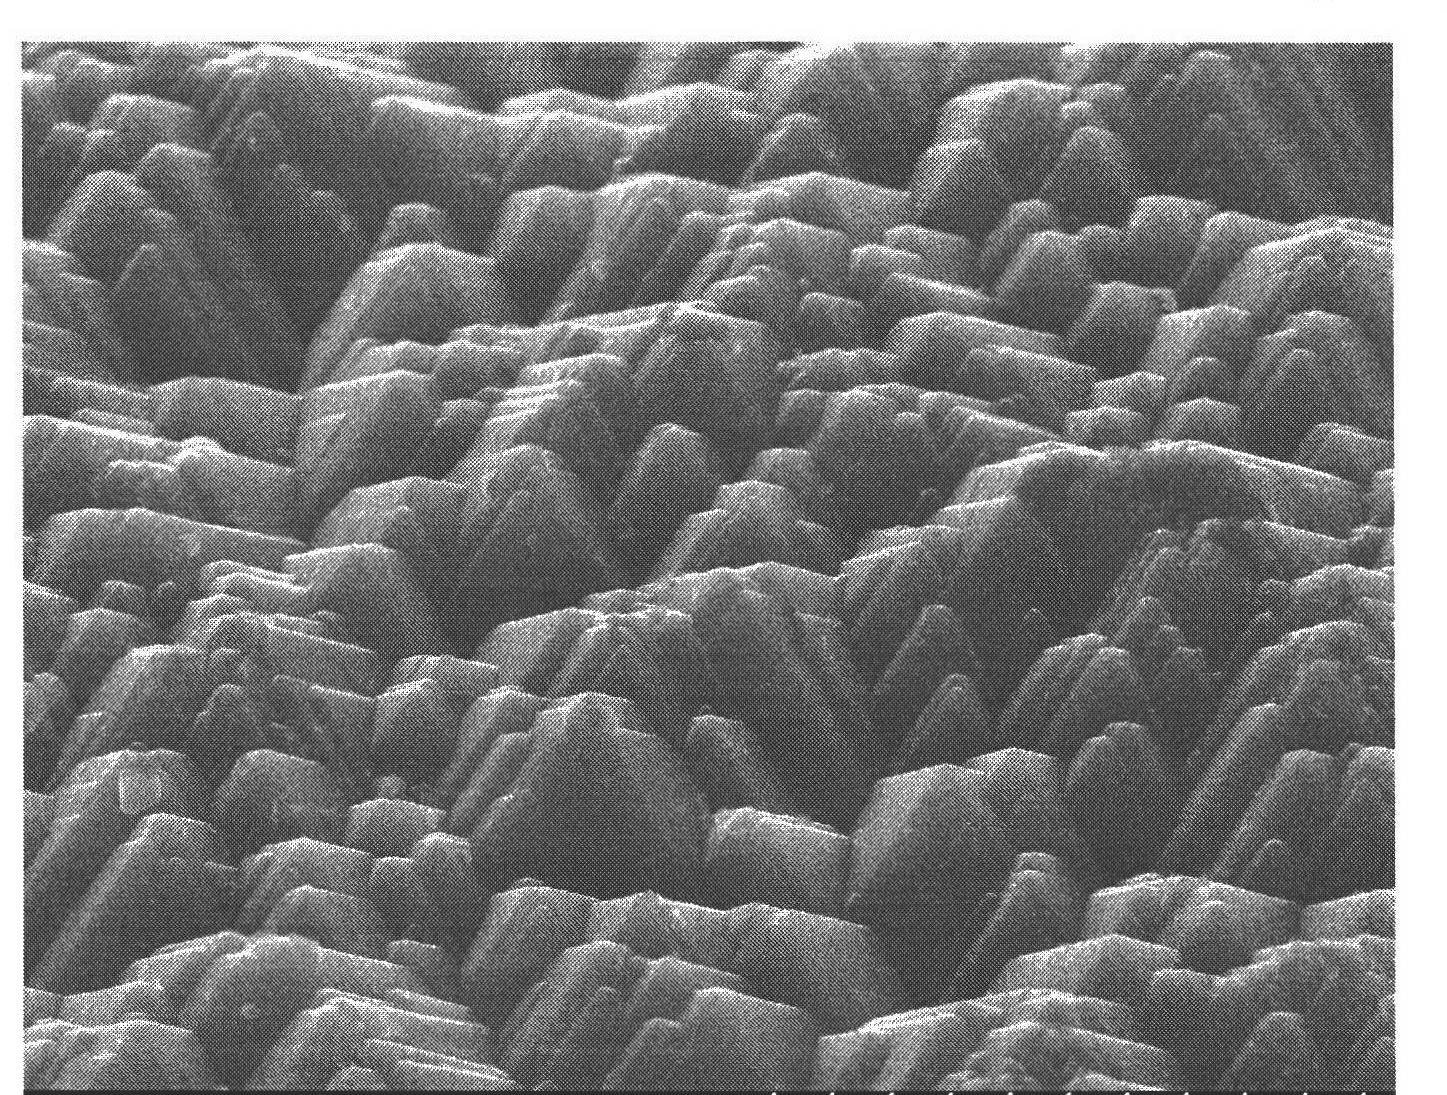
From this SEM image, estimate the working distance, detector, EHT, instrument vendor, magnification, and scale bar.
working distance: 10 mm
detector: SE(U)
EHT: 15 kV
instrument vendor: Hitachi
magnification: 5 K X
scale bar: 10000 nm